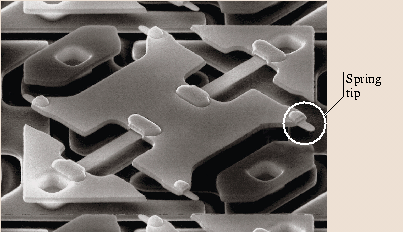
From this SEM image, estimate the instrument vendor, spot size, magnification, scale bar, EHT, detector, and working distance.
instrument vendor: FEI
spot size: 2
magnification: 5 K X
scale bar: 5000 nm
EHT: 10 kV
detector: CDM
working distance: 17 mm